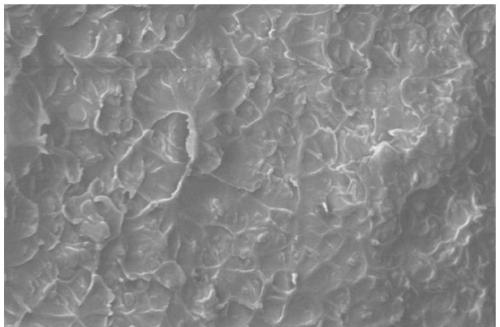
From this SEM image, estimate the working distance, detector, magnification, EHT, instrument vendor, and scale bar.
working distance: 5.9 mm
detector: TLD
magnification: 30 K X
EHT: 15 kV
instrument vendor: FEI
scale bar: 5000 nm